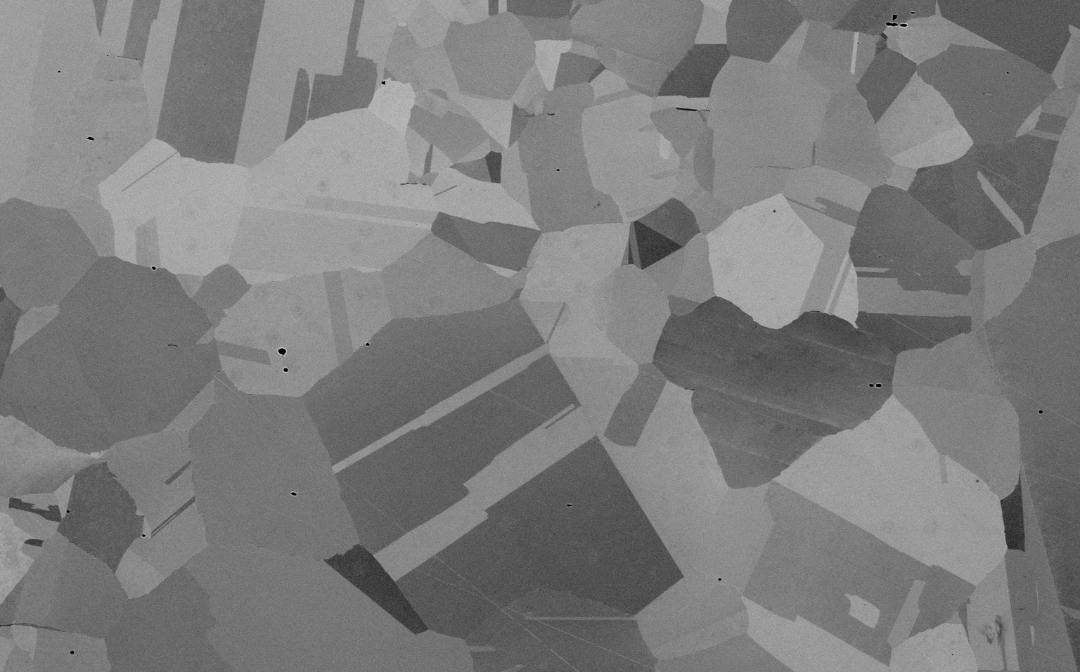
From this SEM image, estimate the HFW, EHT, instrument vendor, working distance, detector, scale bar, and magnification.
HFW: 508 µm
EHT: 20 kV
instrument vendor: other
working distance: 8.863 mm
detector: BSD Full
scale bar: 150000 nm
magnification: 0.25 K X